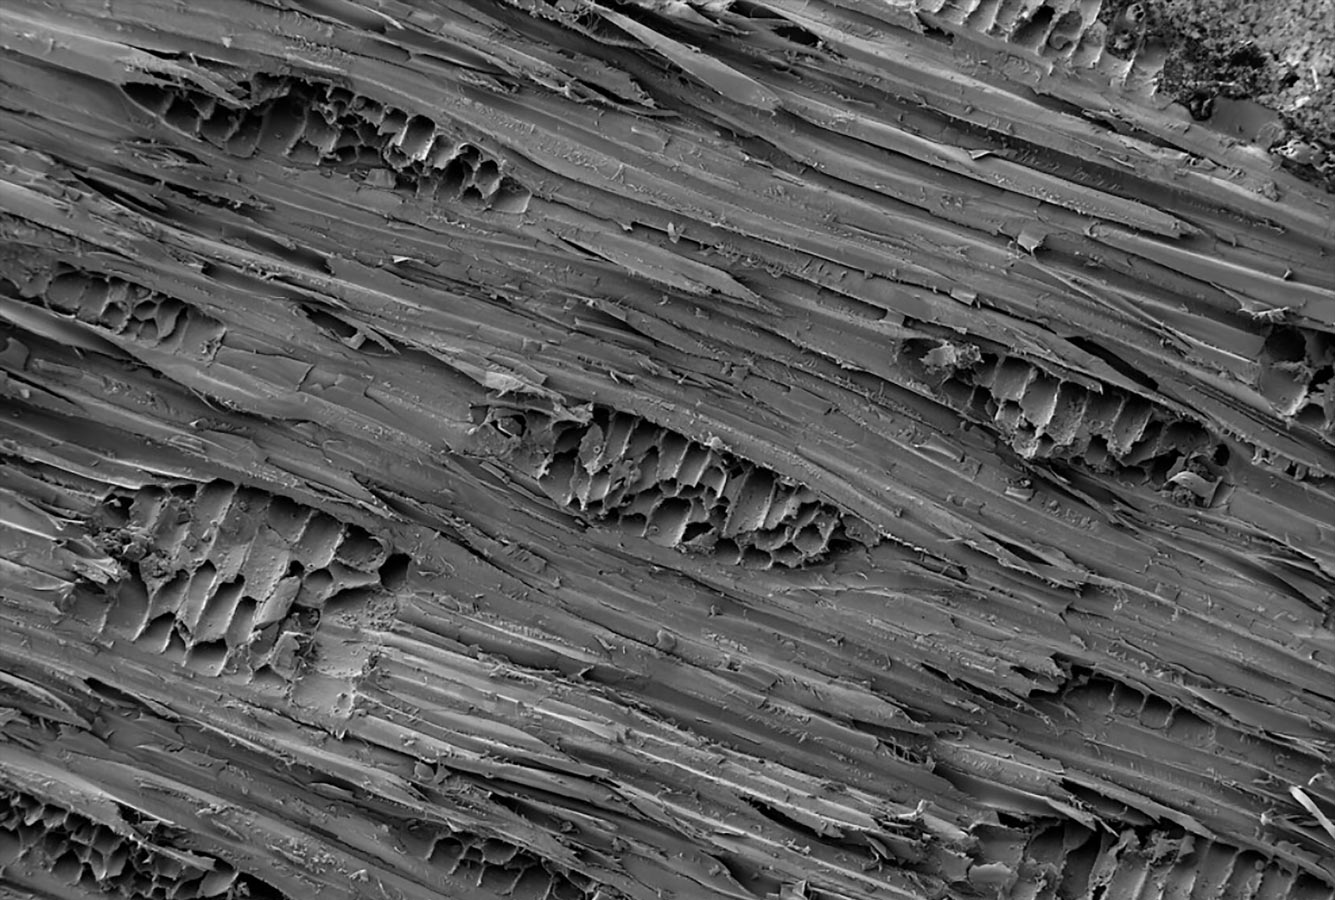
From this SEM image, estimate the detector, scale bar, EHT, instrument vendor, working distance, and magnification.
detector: SE2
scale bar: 100000 nm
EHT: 3 kV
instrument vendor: Zeiss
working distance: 17.6 mm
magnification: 0.1 K X